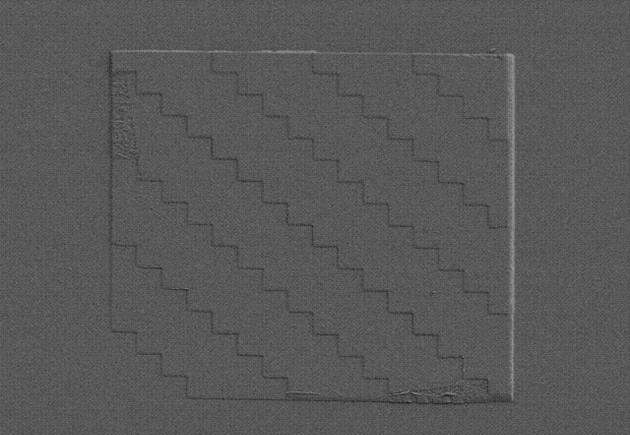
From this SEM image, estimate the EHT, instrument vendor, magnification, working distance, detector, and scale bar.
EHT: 5 kV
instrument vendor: Hitachi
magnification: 1 K X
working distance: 8.8 mm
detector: SE(L)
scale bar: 50000 nm